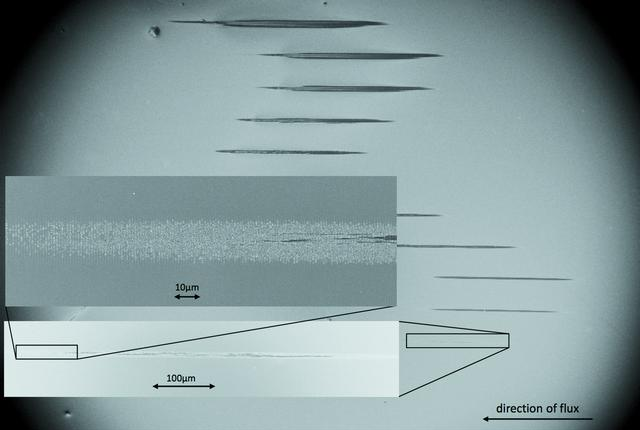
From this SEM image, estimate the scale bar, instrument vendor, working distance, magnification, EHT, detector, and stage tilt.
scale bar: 200000 nm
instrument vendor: Zeiss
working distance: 3.7 mm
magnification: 0.106 K X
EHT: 5 kV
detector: InLens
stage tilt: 0°